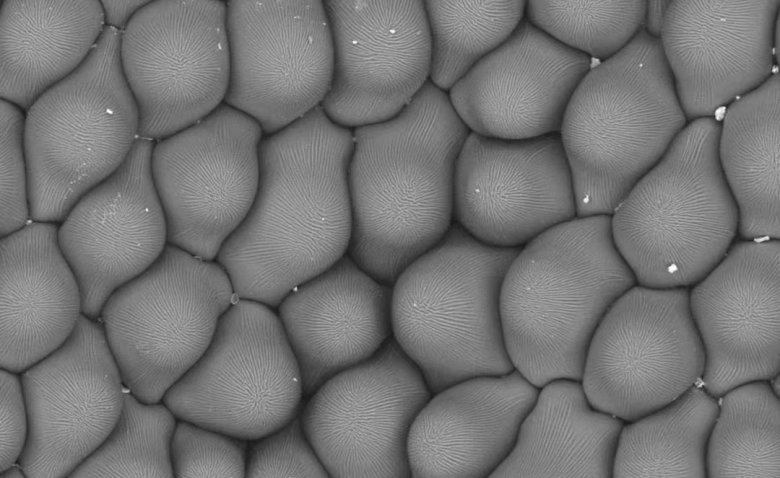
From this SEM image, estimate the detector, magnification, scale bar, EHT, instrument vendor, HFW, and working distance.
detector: BSD Full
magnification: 0.5 K X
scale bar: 80000 nm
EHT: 10 kV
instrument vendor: Thermo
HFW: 254 µm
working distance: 7.613 mm